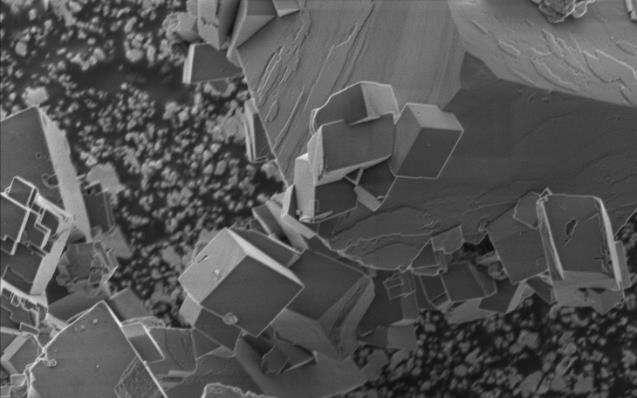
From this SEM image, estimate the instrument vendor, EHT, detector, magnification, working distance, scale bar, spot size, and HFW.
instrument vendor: FEI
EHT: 2 kV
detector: ETD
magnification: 18.102 K X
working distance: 11 mm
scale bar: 5000 nm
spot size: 2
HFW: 22.9 µm